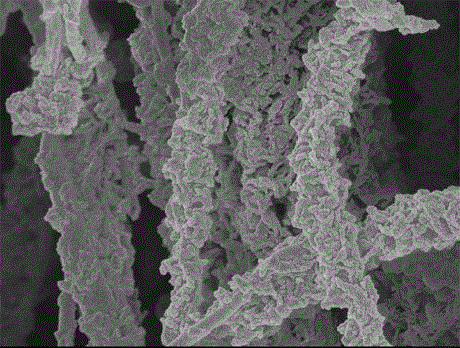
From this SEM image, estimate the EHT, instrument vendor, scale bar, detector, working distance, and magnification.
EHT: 5 kV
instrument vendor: JEOL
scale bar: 1000 nm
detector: SEI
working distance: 7.7 mm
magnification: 20 K X